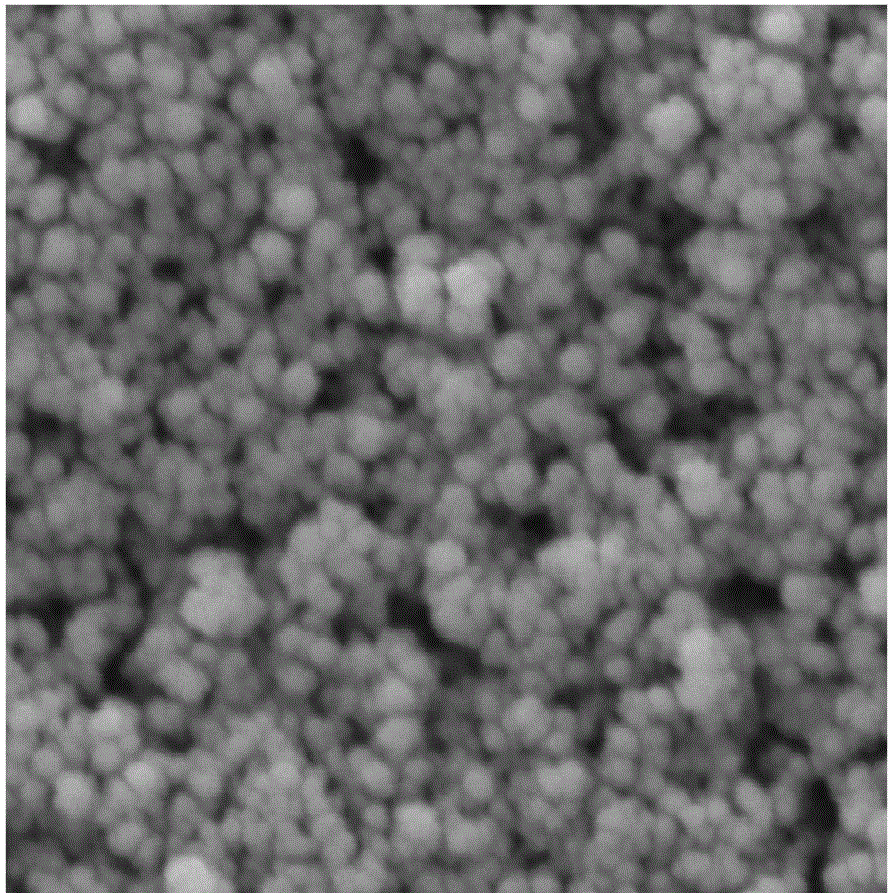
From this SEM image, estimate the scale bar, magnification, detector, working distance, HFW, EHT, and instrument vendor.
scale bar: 200 nm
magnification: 200 K X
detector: SE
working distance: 9.95 mm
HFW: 1.04 µm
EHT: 10 kV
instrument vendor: Tescan